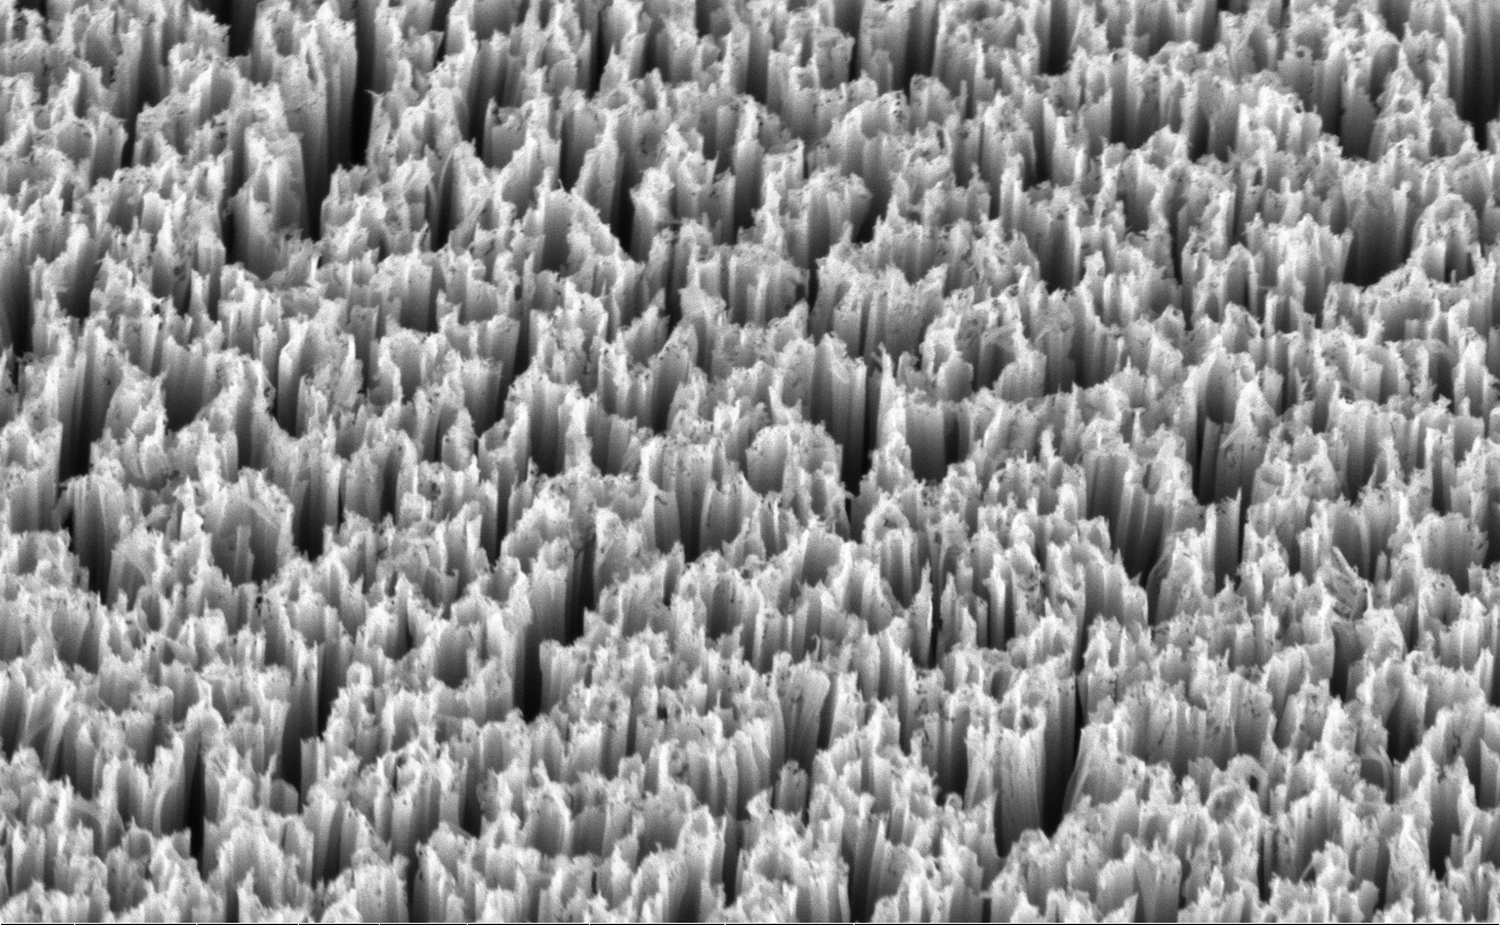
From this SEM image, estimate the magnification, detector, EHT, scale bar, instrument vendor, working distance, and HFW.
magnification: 25 K X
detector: TLD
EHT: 3 kV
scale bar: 1000 nm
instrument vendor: FEI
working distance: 4.3 mm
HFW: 5.08 µm